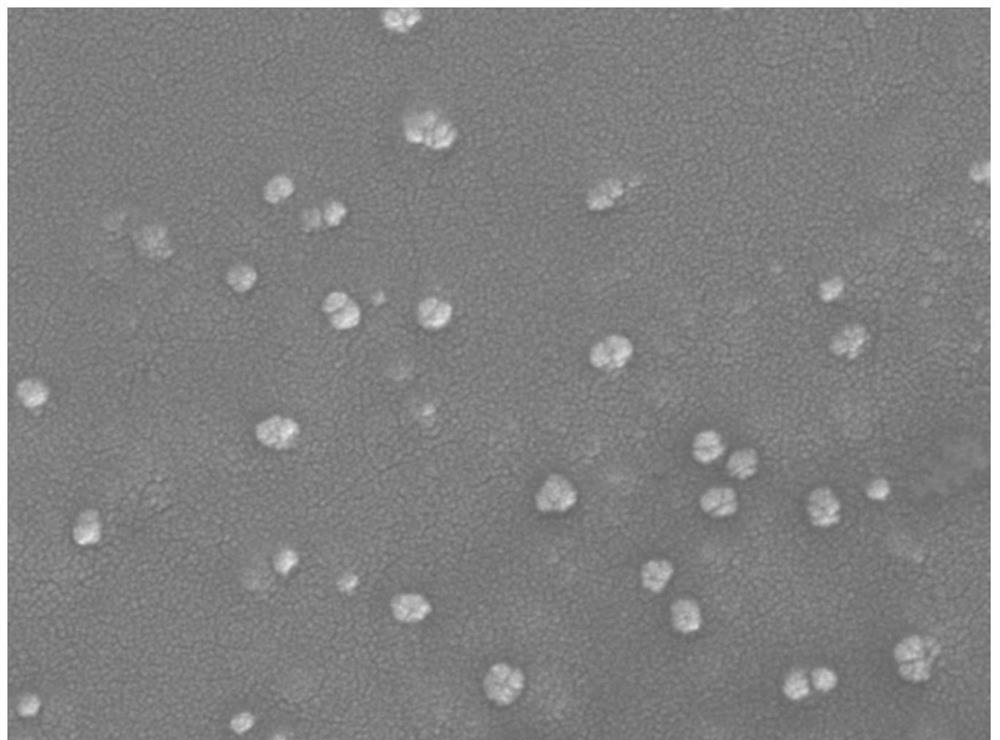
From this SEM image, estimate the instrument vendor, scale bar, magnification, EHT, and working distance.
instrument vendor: JEOL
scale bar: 100 nm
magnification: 50 K X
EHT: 5 kV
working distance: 6.7 mm